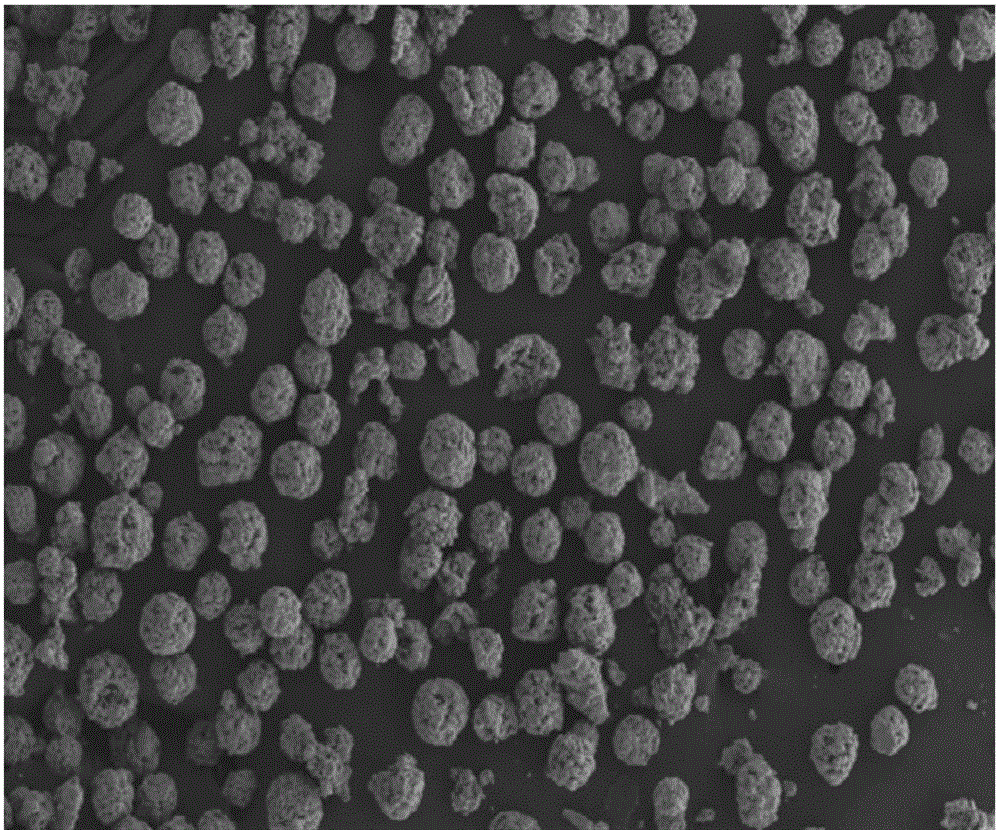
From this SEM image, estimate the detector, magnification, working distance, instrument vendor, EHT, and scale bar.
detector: SE2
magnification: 0.15 K X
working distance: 9.5 mm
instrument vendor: Zeiss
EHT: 15 kV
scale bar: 100000 nm